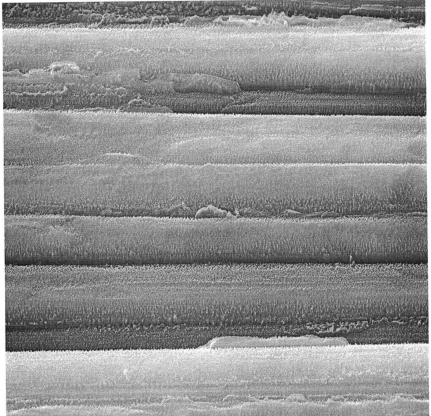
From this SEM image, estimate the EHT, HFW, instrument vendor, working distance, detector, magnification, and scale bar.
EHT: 30 kV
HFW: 43 µm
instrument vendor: Tescan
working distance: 15.06 mm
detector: SE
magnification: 6.72 K X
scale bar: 10000 nm